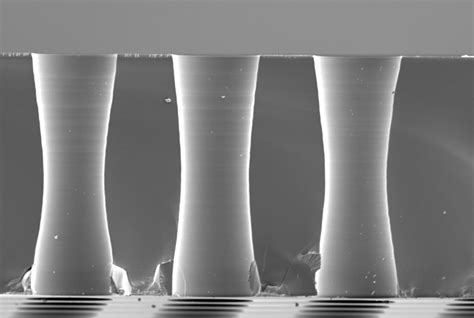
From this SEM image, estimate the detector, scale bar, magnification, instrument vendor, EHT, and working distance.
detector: SEI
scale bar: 10000 nm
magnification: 1 K X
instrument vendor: JEOL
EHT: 5 kV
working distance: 23 mm